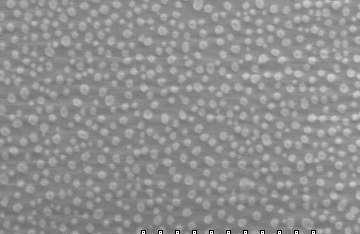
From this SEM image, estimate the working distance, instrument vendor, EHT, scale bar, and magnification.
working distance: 15.4 mm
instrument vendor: Hitachi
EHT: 5 kV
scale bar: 5000 nm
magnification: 7 K X